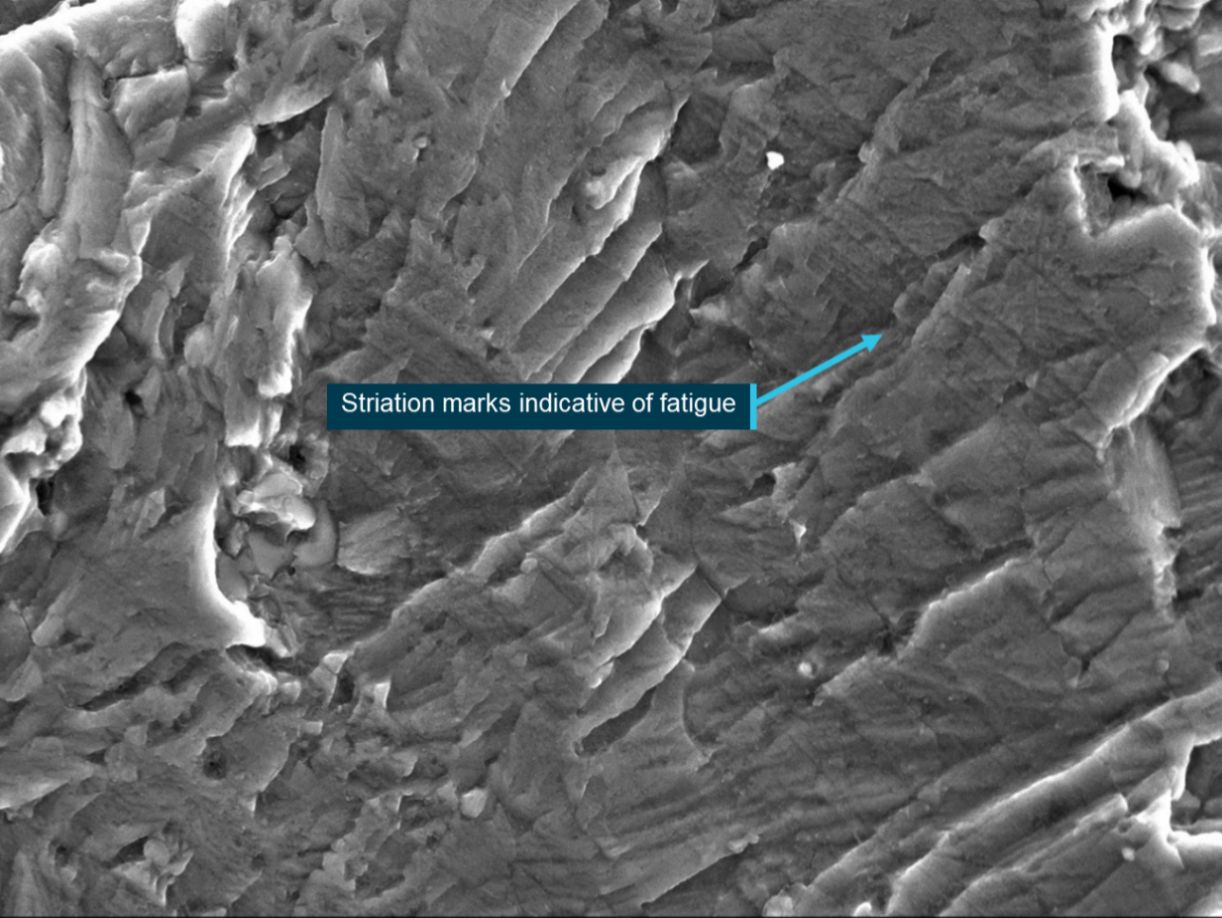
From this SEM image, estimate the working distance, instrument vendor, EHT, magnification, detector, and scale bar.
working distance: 13 mm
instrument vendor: JEOL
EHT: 20 kV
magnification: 1.2 K X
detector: SED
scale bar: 10000 nm